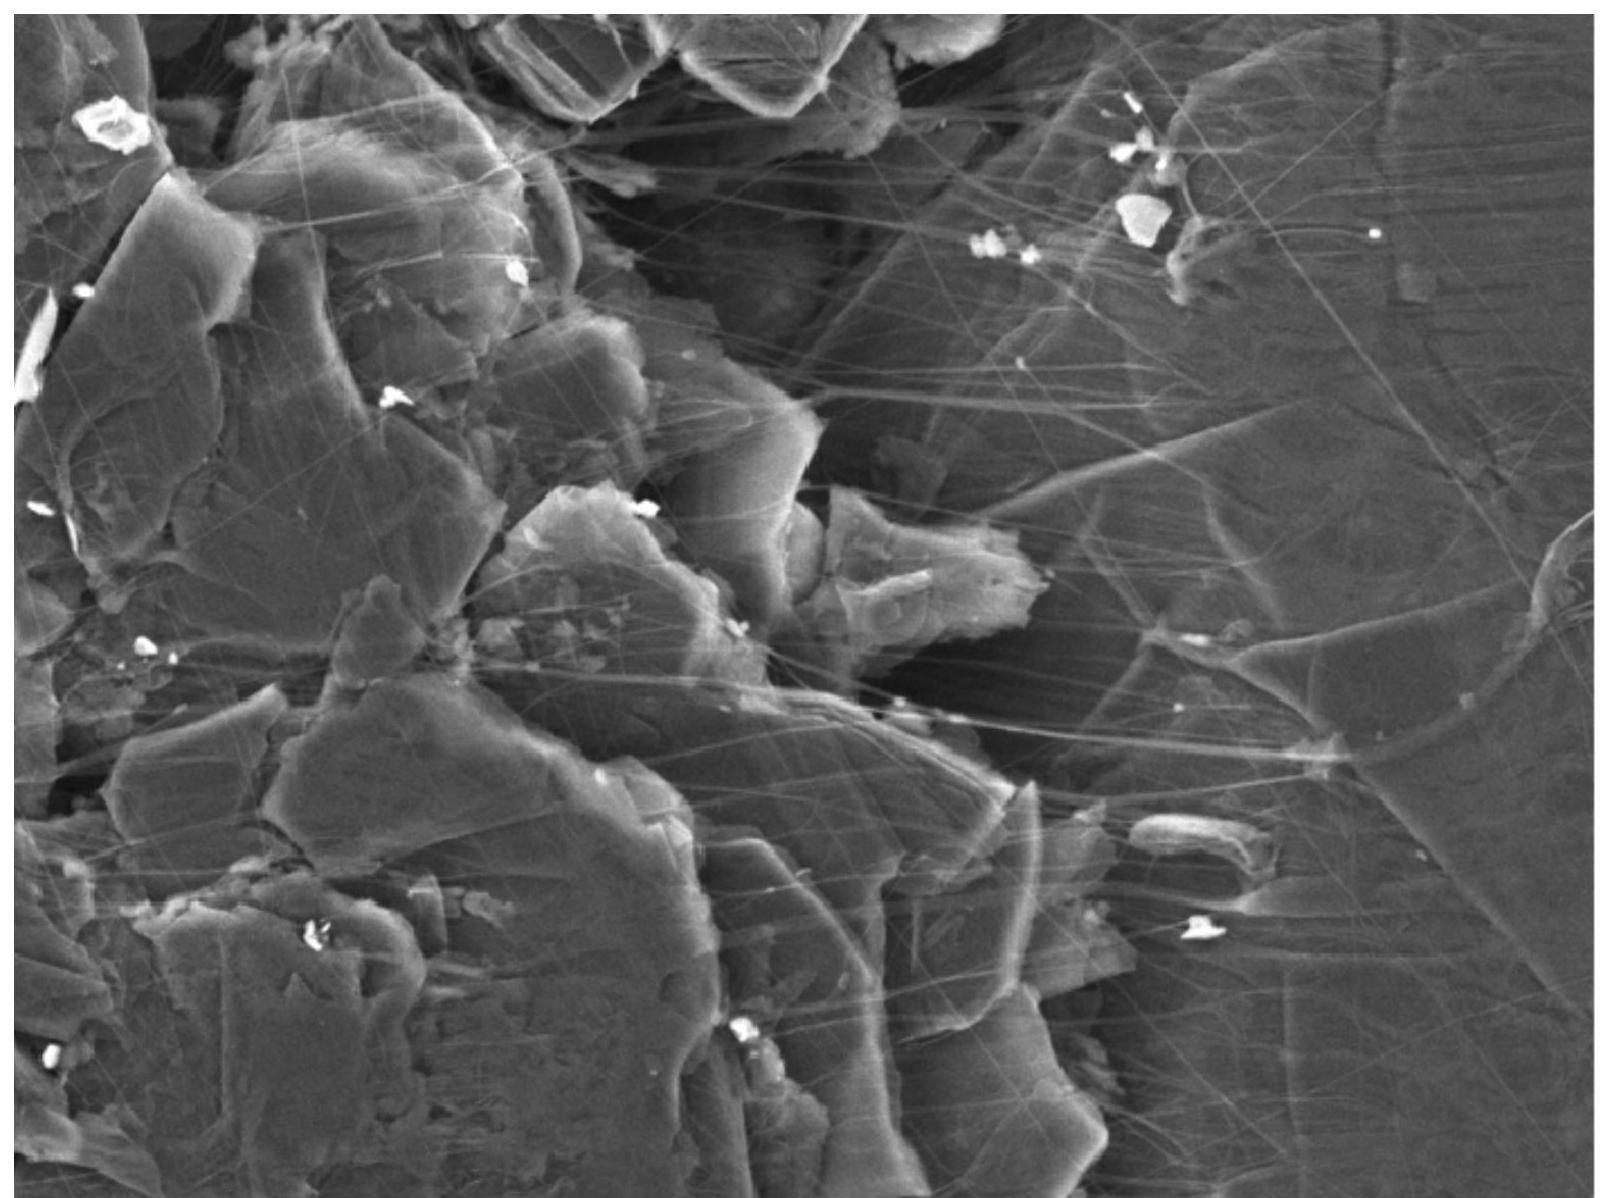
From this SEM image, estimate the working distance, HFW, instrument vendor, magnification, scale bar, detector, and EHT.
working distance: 7.02 mm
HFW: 25.3 µm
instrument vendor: Tescan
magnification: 10 K X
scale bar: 5000 nm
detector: SE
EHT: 5 kV